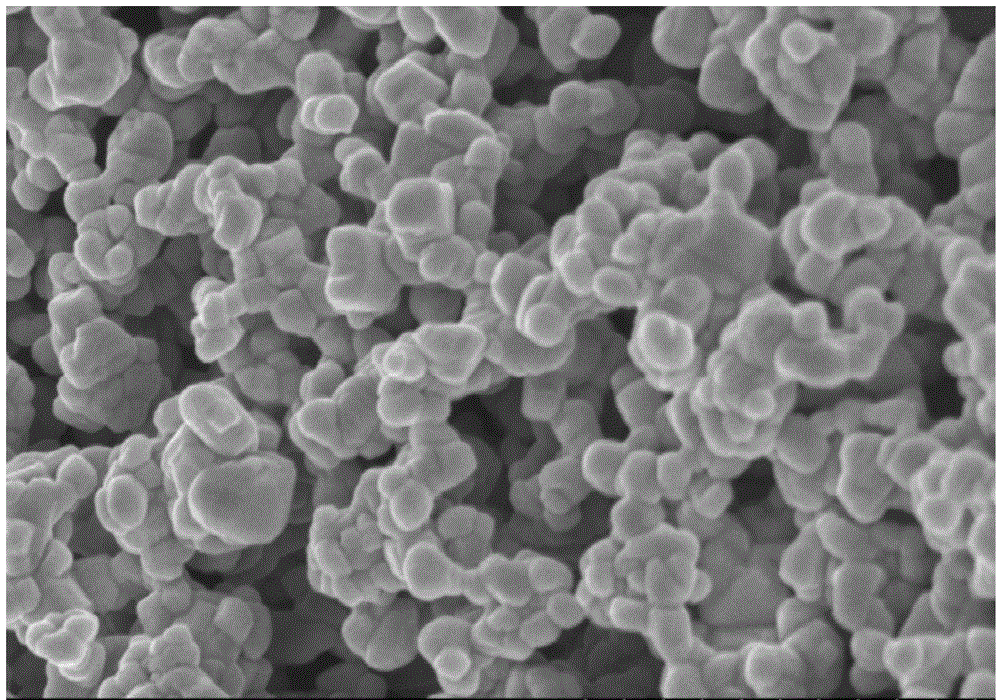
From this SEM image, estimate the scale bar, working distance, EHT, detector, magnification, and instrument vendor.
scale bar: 1000 nm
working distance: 9.4 mm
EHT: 3 kV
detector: SE(M)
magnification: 50 K X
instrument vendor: Hitachi